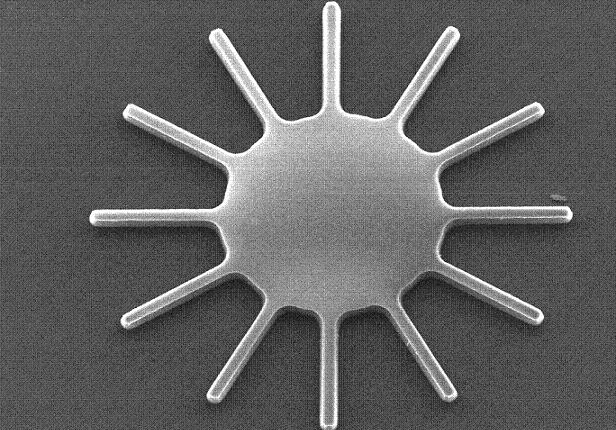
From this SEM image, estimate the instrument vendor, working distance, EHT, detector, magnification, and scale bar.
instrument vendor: Hitachi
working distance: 8 mm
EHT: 10 kV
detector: SE(M)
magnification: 0.8 K X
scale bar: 50000 nm